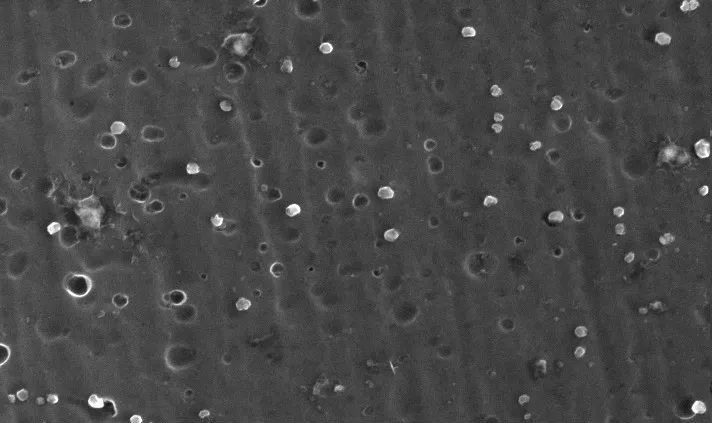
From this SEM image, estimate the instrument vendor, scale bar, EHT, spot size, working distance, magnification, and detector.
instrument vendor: FEI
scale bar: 1000 nm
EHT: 20 kV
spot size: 4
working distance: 7.5 mm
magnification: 50 K X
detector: SE